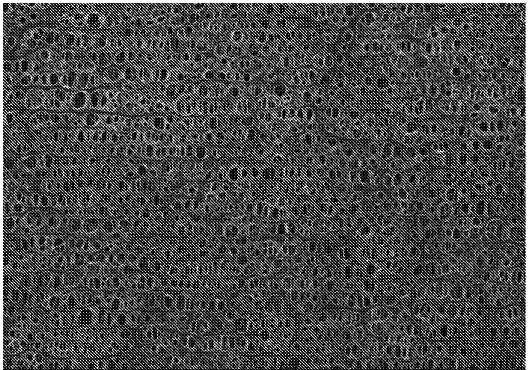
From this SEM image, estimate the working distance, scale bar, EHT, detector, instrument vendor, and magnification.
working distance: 4.8 mm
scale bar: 5000 nm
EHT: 1 kV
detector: SE(U)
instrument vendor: Hitachi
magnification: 10 K X